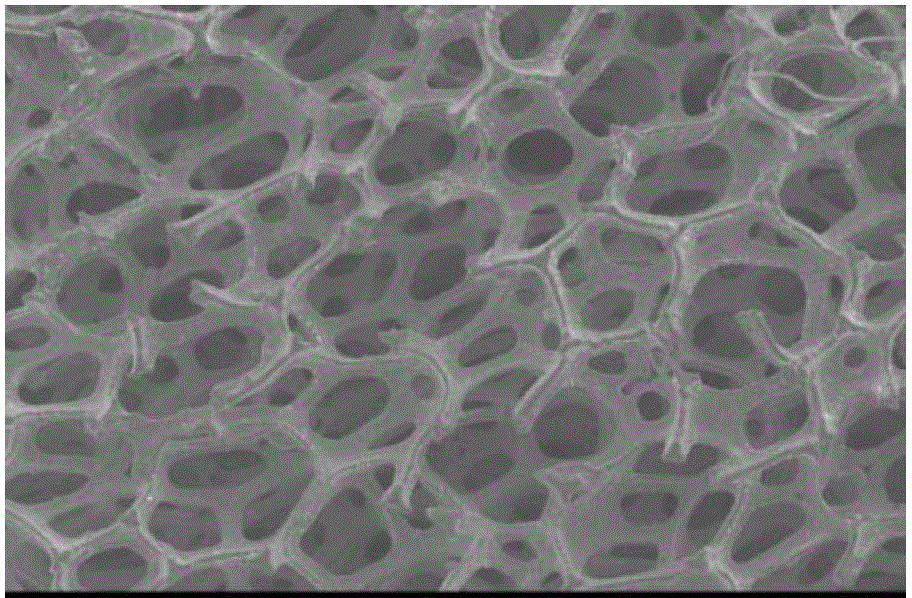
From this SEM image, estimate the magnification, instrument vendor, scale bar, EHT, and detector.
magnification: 0.05 K X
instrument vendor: JEOL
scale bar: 500000 nm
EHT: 20 kV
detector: SEI